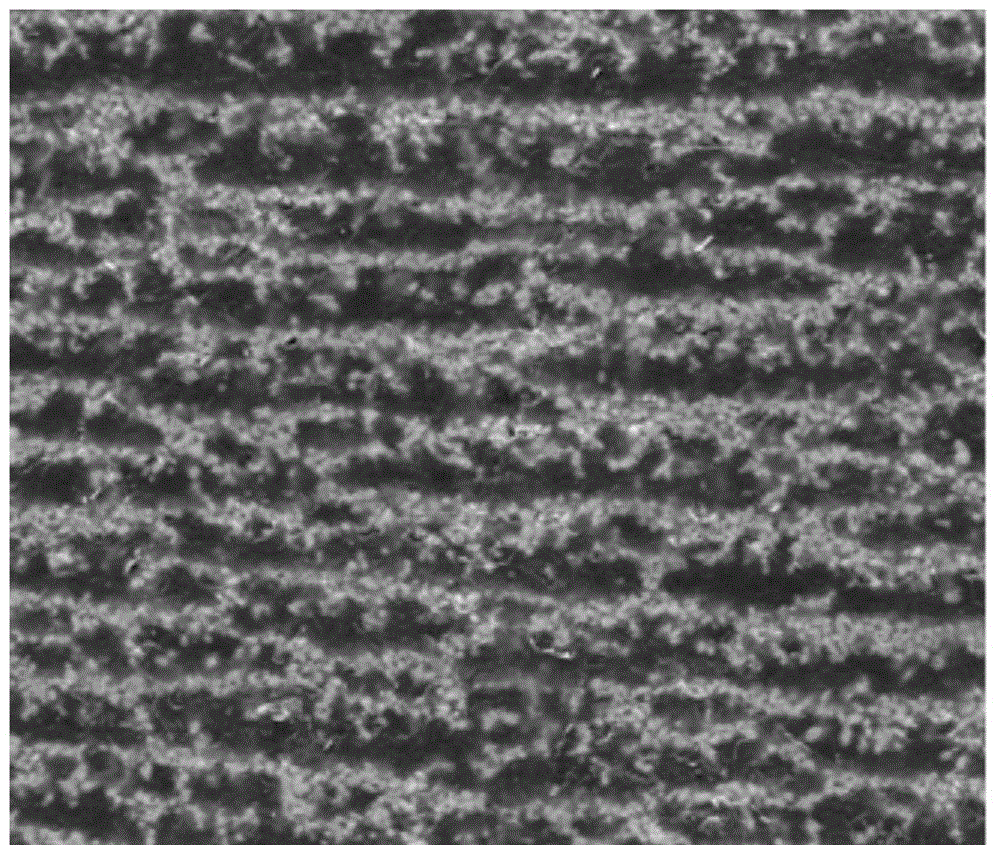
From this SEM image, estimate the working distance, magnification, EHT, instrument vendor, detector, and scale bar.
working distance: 10.2 mm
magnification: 2 K X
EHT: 30 kV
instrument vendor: FEI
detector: SE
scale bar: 50000 nm